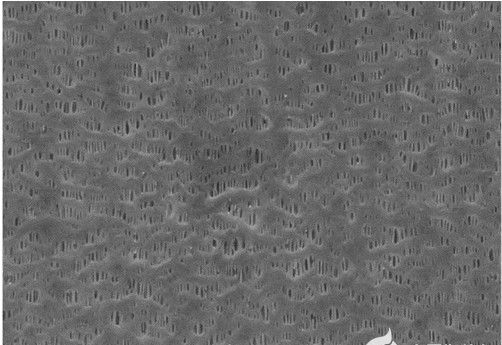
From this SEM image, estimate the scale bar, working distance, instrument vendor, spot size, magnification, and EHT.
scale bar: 1000 nm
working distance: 5 mm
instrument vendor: JEOL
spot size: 7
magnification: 30 K X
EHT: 20 kV